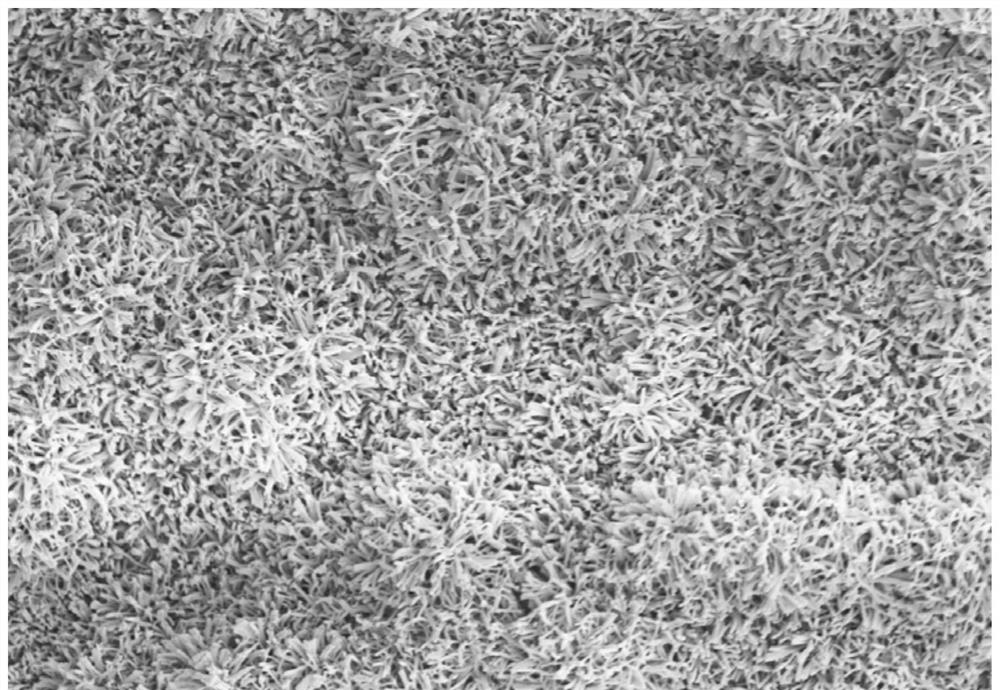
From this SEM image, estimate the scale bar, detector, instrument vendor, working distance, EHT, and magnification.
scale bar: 20000 nm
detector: SE(M,LA0)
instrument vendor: Hitachi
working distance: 12 mm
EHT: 5 kV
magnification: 2 K X